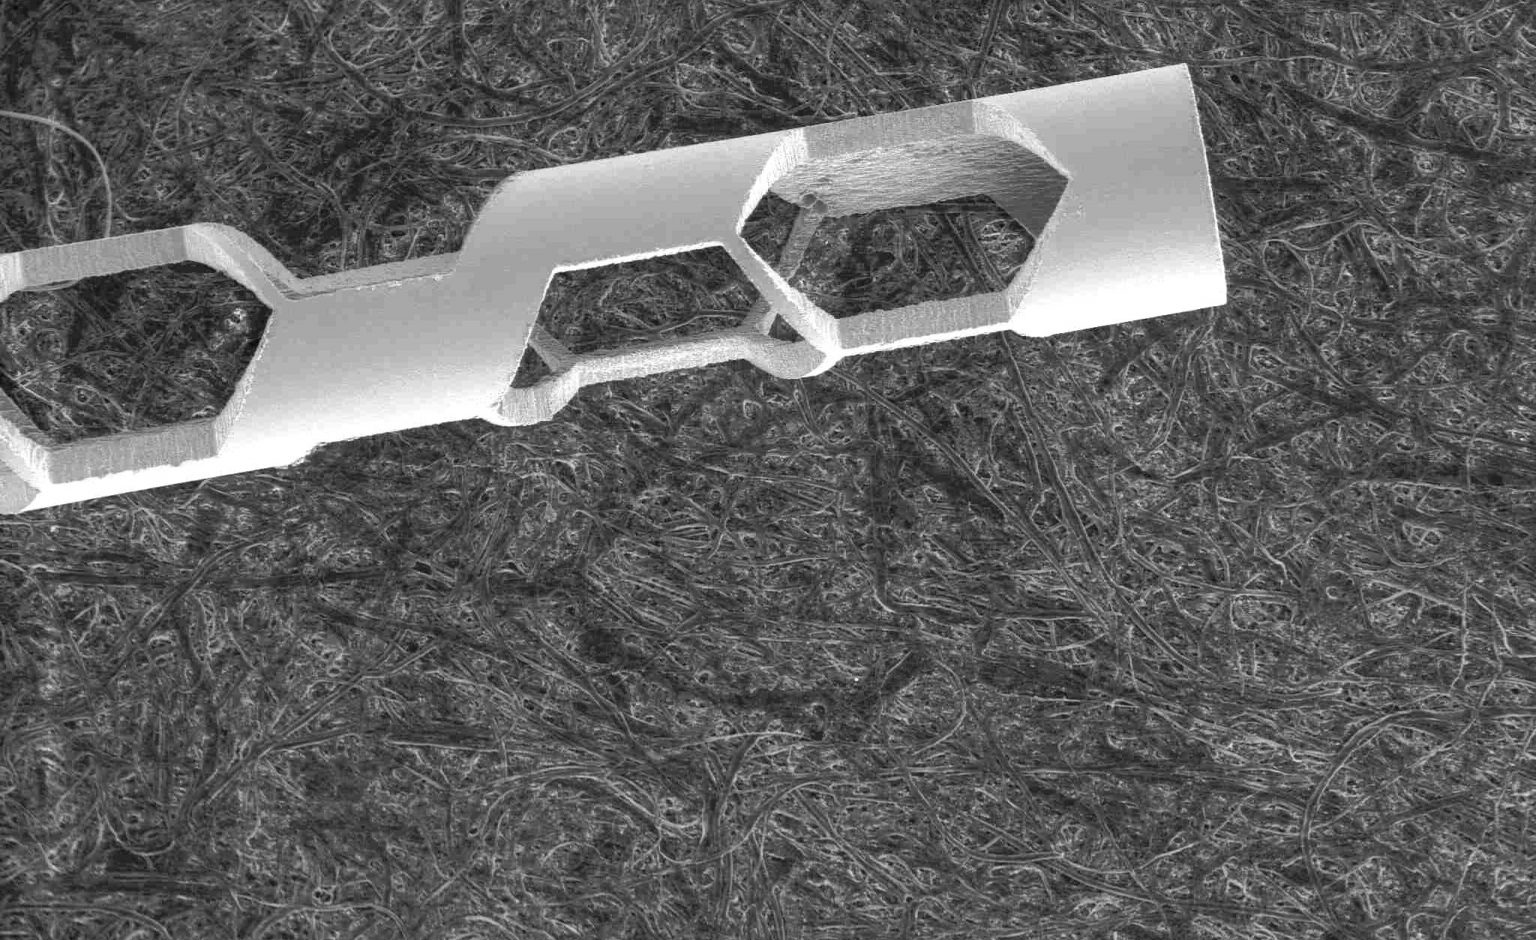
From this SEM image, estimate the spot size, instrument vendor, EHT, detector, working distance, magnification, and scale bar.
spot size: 3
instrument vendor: FEI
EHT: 30 kV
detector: LFD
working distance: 23.5 mm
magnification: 0.05 K X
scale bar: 1000000 nm